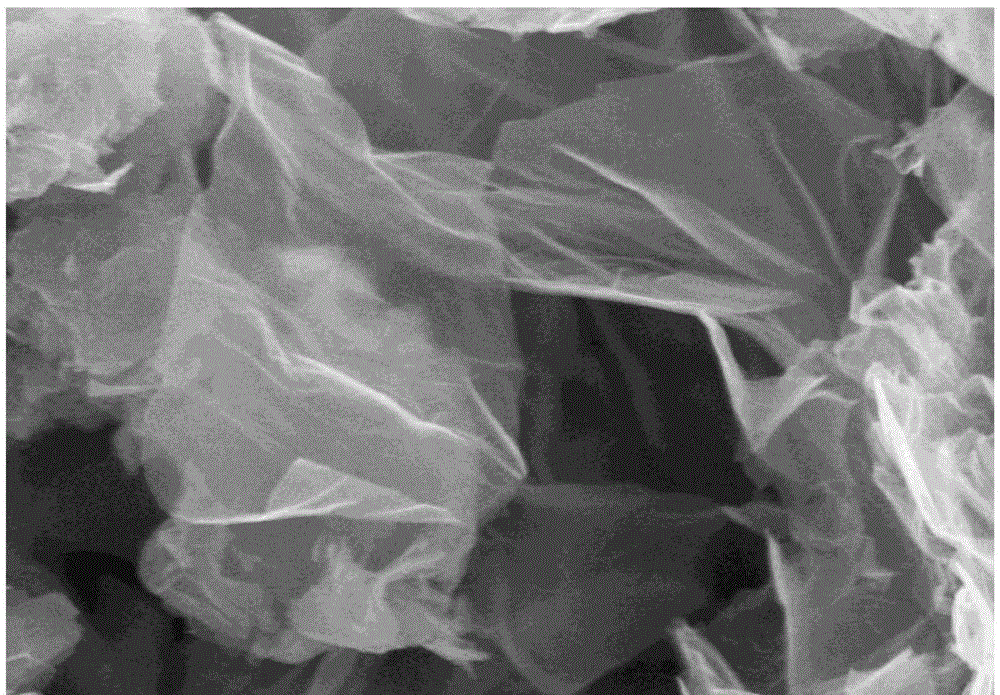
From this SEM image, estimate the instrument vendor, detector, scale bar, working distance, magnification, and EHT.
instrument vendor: Hitachi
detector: SE(M)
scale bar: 1000 nm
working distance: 9.5 mm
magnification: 45 K X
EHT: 5 kV